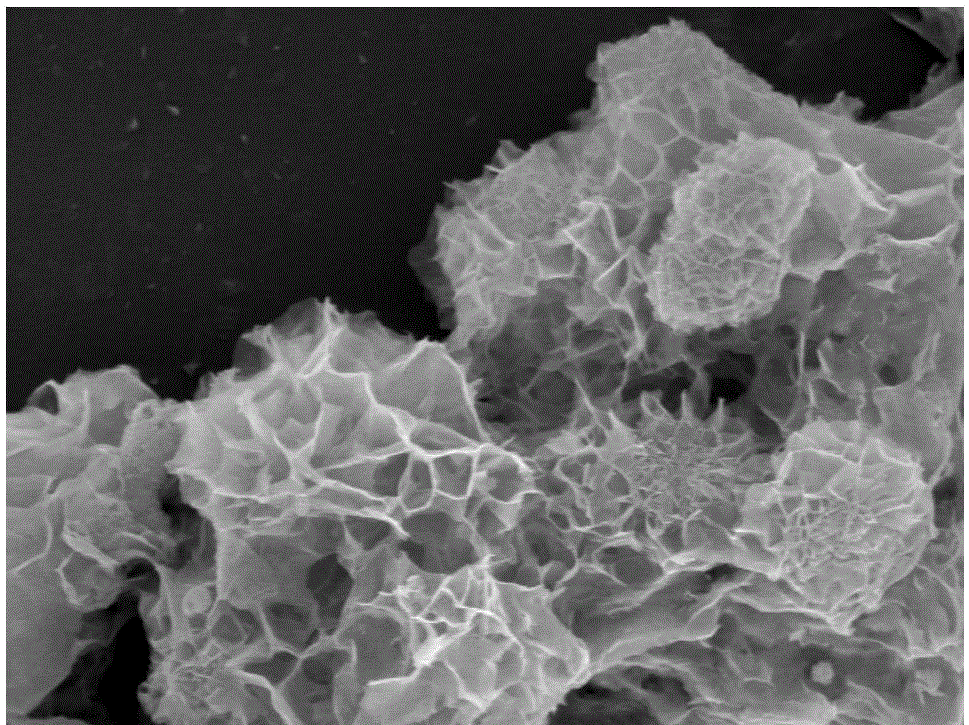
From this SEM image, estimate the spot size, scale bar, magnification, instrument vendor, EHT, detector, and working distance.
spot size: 5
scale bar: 2000 nm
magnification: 10 K X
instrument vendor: FEI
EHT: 10 kV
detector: TLD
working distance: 5 mm